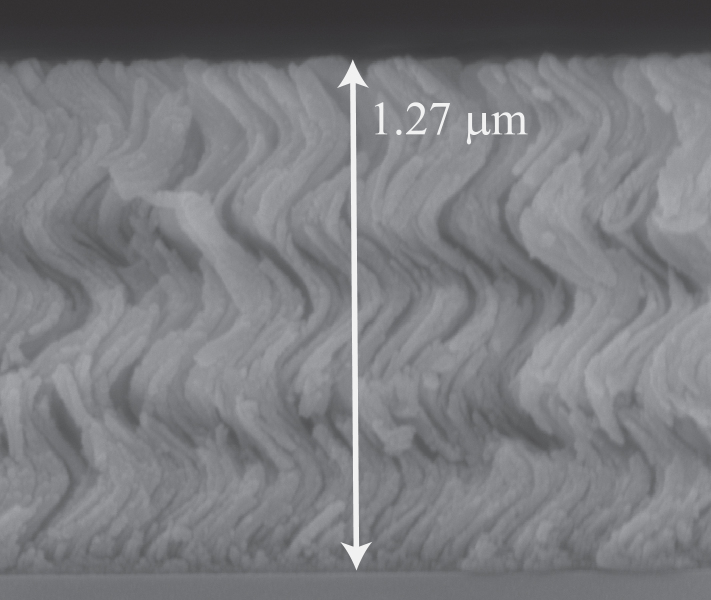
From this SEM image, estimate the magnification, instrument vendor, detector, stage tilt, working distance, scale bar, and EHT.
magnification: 159.969 K X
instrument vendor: FEI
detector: TLD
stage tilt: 0°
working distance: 3.1 mm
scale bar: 500 nm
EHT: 5 kV